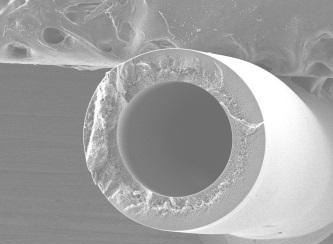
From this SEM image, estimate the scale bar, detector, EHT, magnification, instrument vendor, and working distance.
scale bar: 100000 nm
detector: LEI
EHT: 10 kV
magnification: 0.075 K X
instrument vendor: JEOL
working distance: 7.9 mm